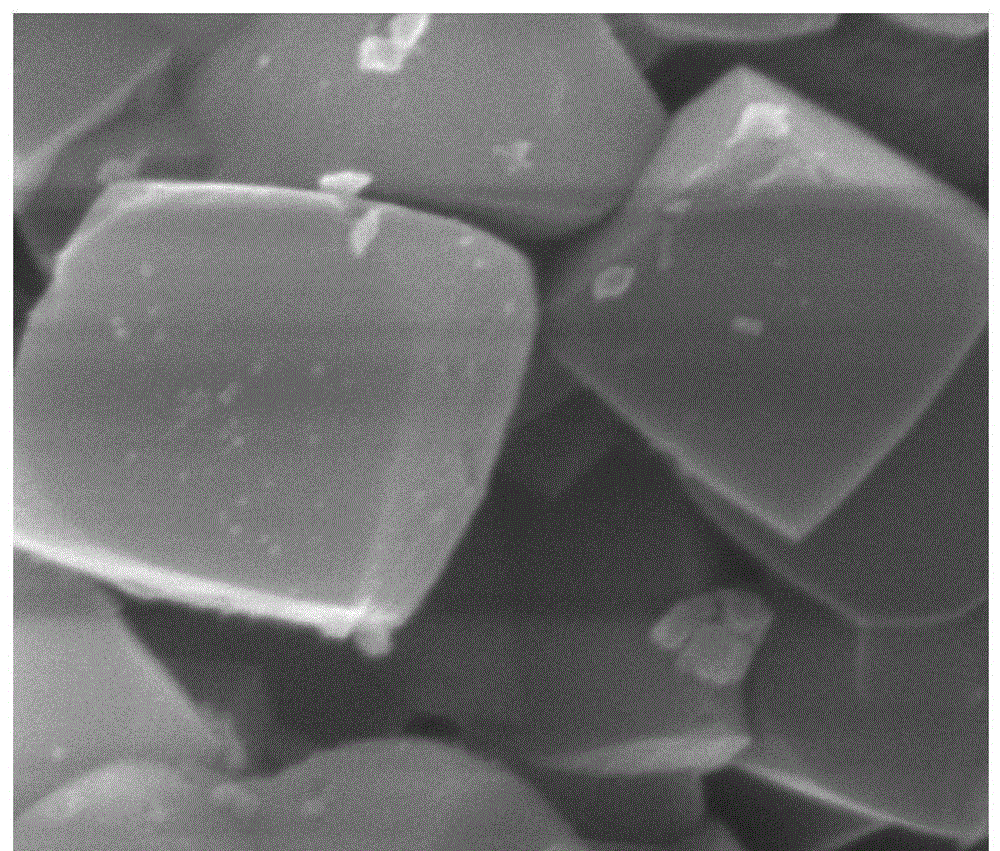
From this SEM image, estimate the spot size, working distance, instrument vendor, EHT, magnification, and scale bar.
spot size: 2.5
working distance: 10.7 mm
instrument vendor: FEI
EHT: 20 kV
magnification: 100 K X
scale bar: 1000 nm